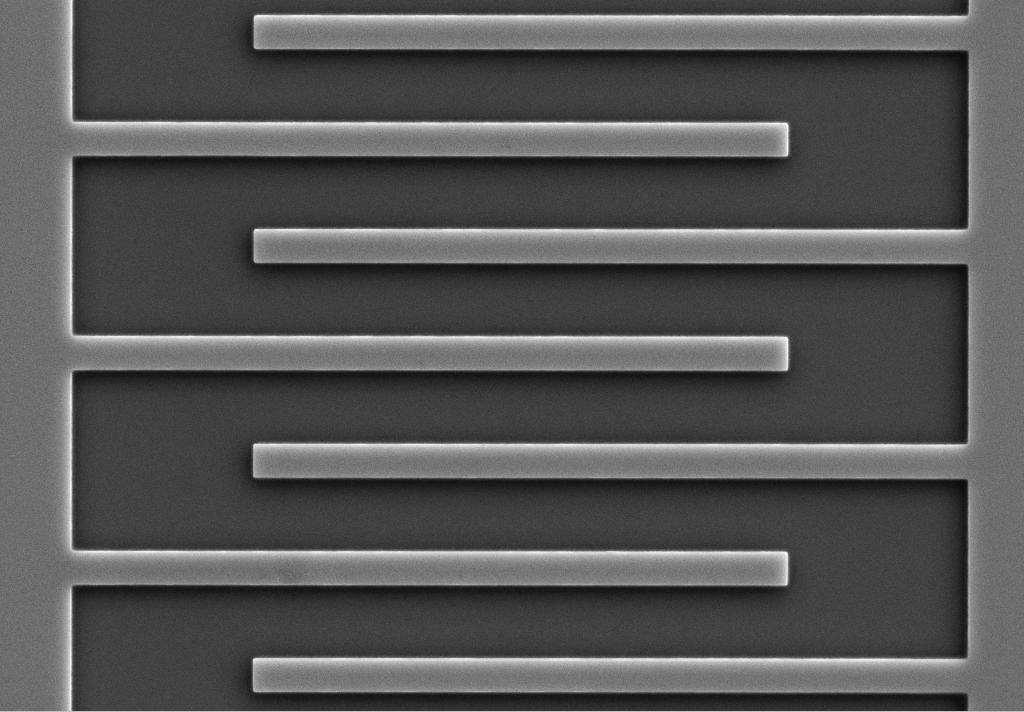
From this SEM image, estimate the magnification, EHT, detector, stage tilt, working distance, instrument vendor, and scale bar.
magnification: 4 K X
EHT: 10 kV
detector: SE2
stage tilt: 0°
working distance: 12.2 mm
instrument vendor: Zeiss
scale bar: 2000 nm